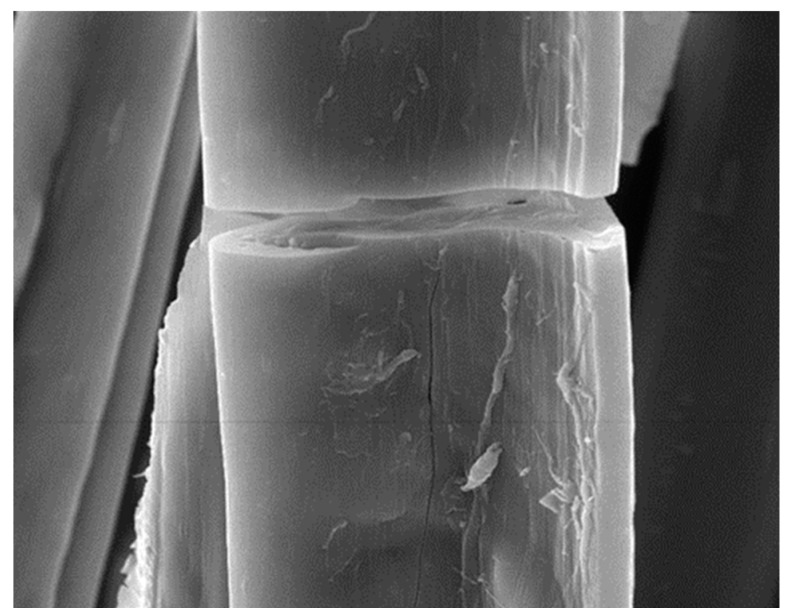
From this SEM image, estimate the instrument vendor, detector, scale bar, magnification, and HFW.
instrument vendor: Tescan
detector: SE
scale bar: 10000 nm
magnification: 5 K X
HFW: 43.3 µm